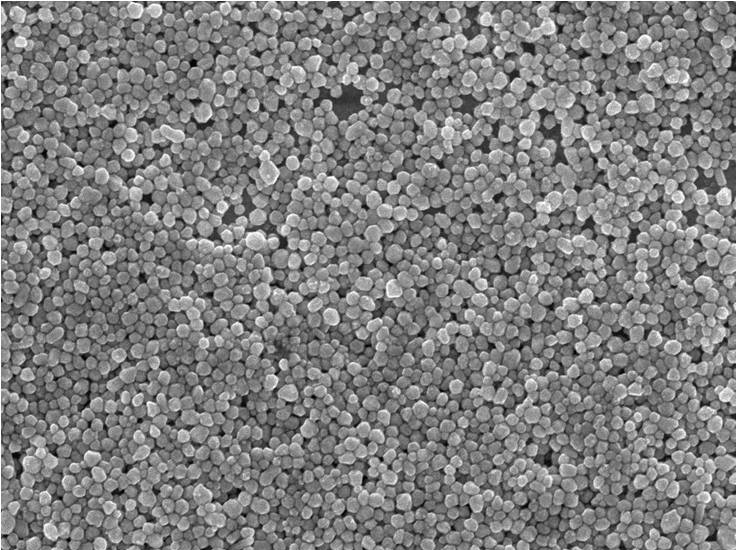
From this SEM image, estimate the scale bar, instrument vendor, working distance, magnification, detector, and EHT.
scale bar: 1000 nm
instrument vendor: JEOL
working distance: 5.9 mm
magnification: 20 K X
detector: SEI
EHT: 10 kV